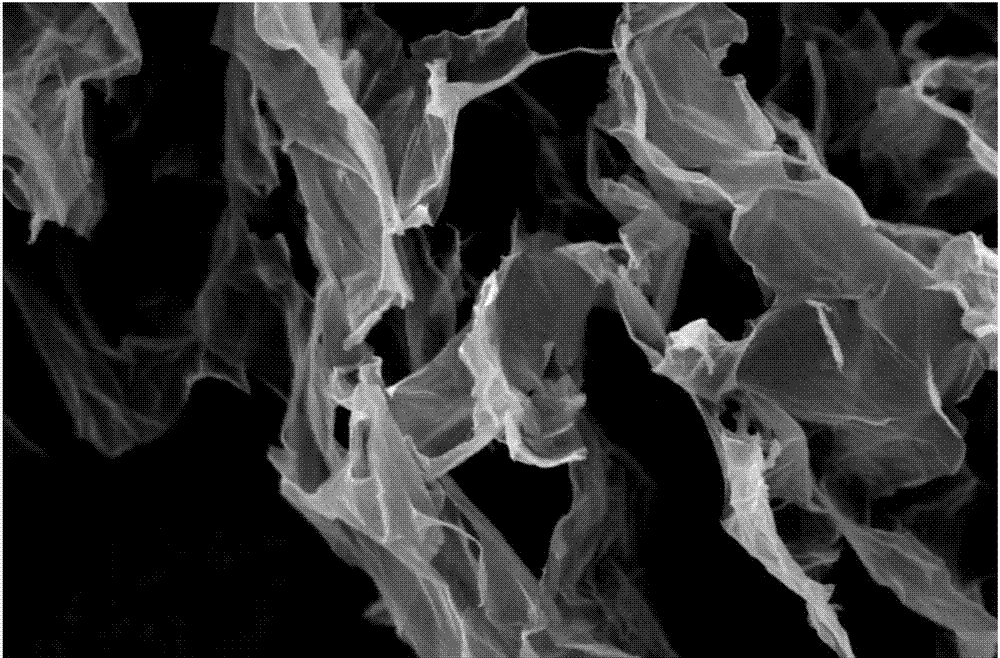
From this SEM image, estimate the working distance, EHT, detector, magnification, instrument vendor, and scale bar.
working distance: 8 mm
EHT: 5 kV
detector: SE1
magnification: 5 K X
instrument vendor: Zeiss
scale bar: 1000 nm